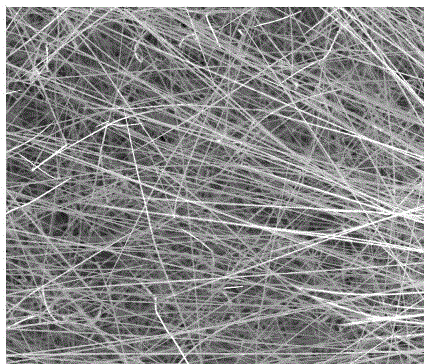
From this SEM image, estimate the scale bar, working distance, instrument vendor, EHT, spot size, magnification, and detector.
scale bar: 10000 nm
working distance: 4.9 mm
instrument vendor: FEI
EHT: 20 kV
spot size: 4.5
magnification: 5 K X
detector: ETD